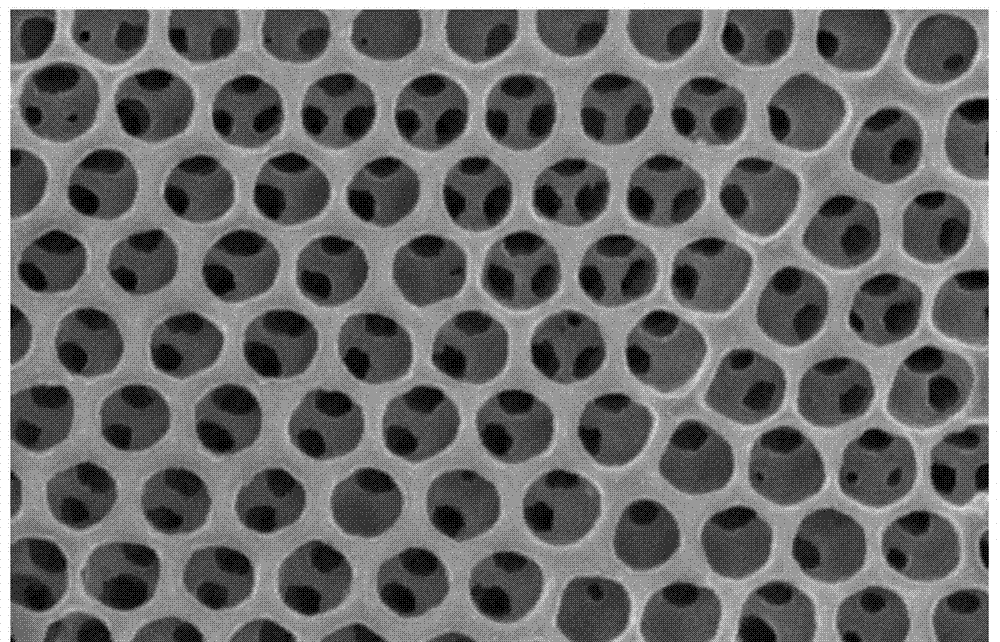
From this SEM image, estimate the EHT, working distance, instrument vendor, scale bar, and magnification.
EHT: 10 kV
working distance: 8.2 mm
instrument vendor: Hitachi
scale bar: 1000 nm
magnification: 35.1 K X